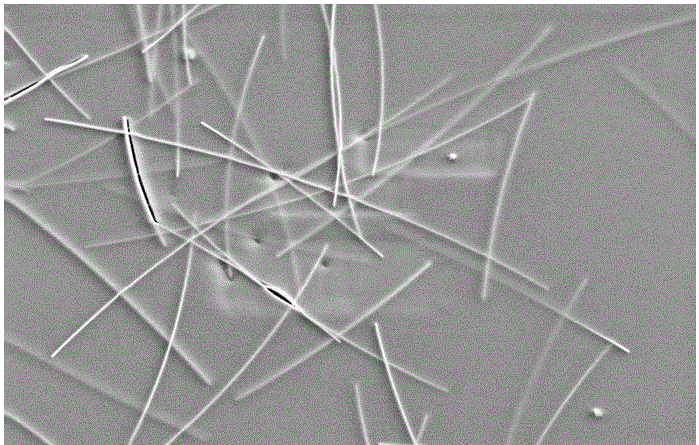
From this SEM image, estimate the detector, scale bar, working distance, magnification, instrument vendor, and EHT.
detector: SE2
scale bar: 1000 nm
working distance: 10.6 mm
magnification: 20 K X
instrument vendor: Zeiss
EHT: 5 kV